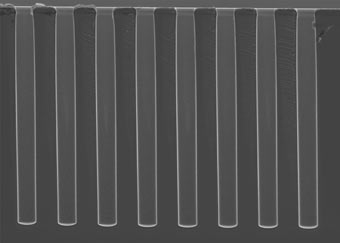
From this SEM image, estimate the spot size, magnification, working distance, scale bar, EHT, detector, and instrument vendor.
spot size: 3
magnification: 1.464 K X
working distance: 4.9 mm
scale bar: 20000 nm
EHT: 5 kV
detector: SE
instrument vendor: FEI